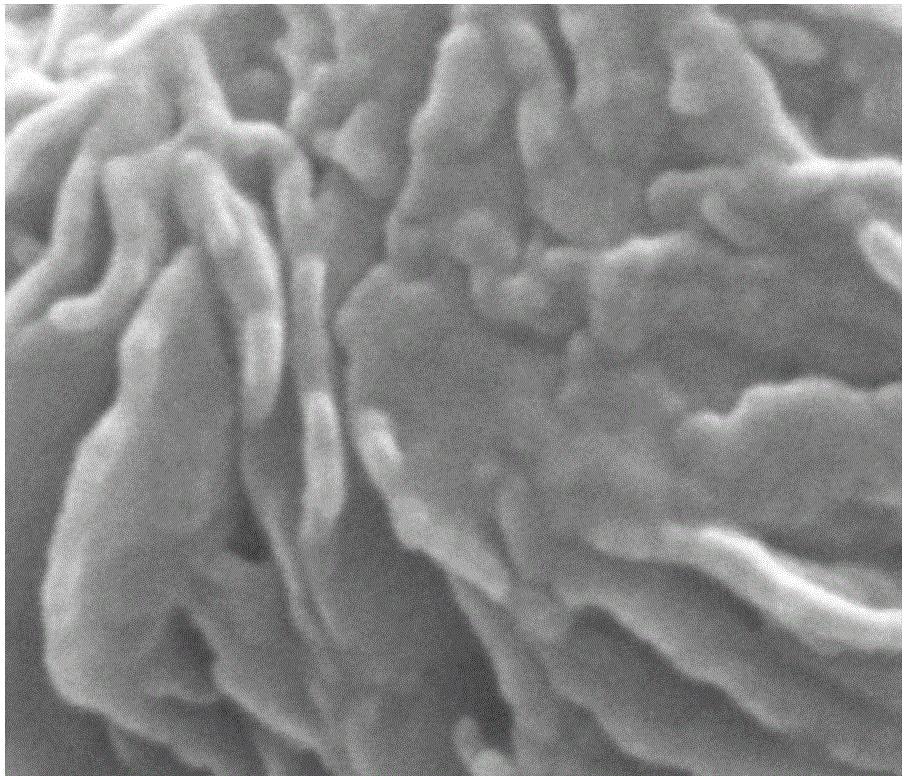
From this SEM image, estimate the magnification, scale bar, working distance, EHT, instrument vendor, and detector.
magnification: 250 K X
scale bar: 100 nm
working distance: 4.7 mm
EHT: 20 kV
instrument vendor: FEI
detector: SE